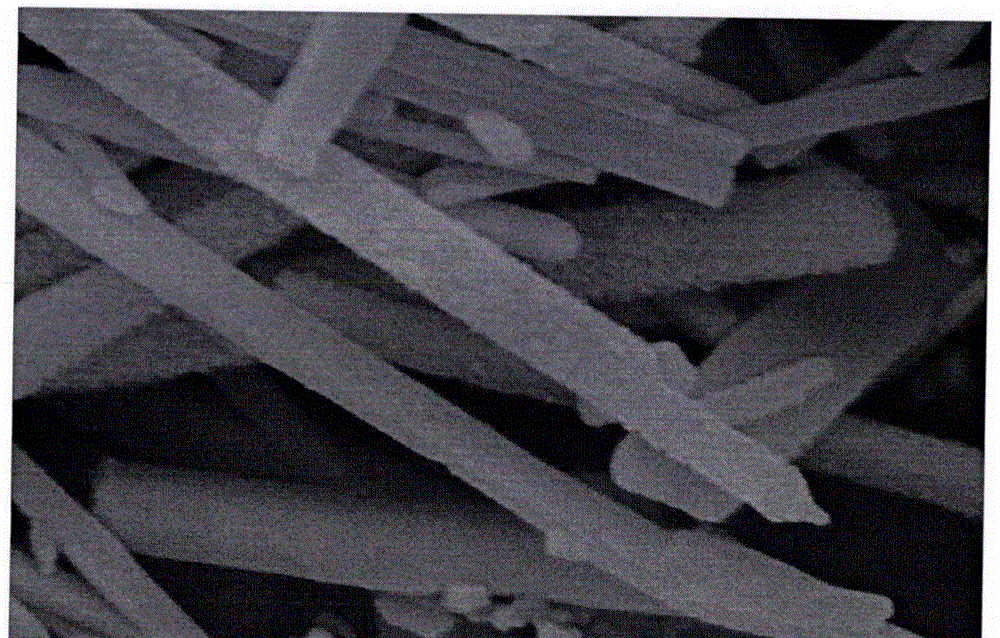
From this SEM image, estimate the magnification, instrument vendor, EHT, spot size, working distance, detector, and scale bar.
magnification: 40 K X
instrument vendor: FEI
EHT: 10 kV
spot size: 3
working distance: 6 mm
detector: SE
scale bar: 500 nm